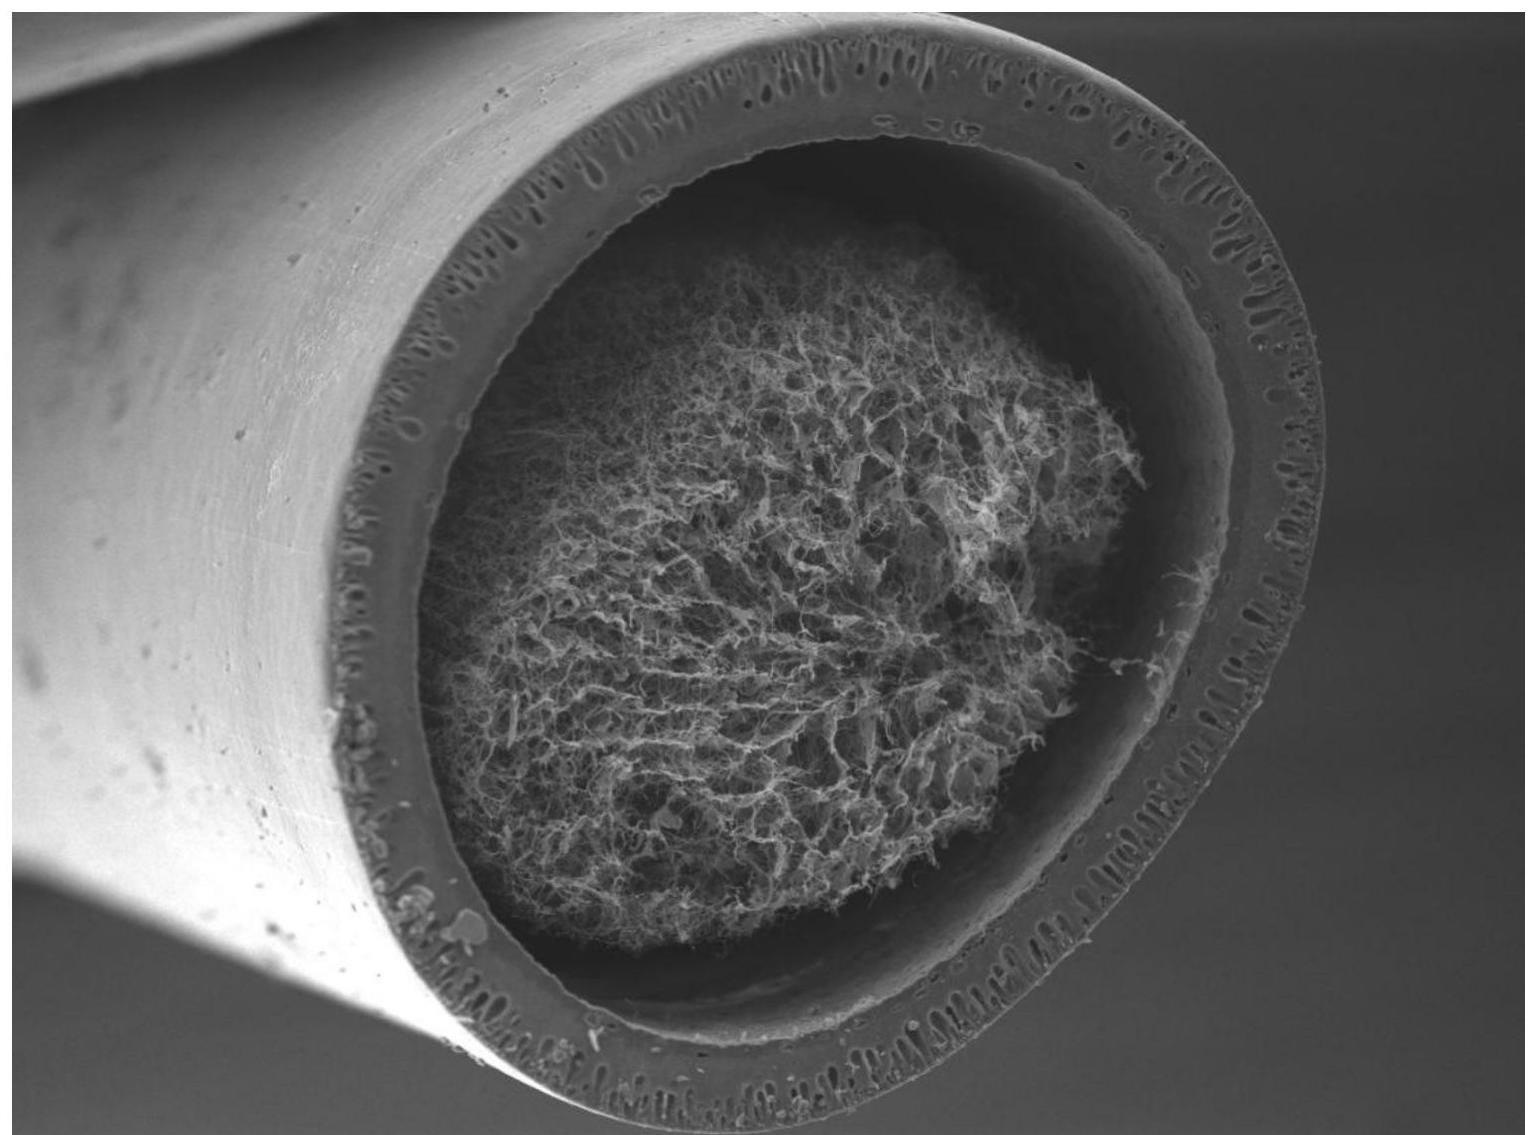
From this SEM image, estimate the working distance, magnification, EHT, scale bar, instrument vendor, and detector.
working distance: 12.1 mm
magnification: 0.075 K X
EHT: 15 kV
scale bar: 200000 nm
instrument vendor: JEOL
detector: SED